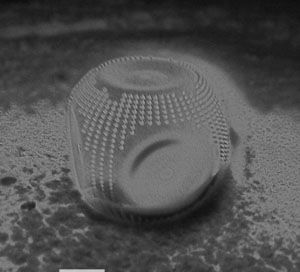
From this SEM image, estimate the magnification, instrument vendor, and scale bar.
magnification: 0.8 K X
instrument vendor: JEOL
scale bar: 10000 nm